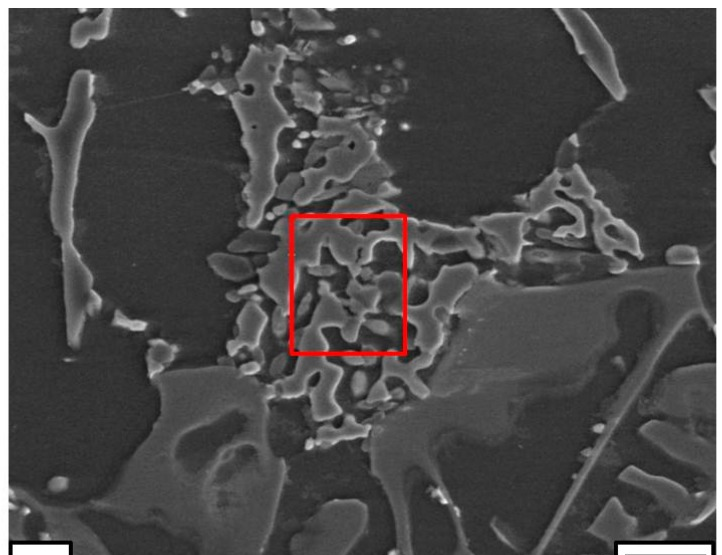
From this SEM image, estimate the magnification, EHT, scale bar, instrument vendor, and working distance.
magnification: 0.858 K X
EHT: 20 kV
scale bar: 15000 nm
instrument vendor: Tescan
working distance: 10.1 mm